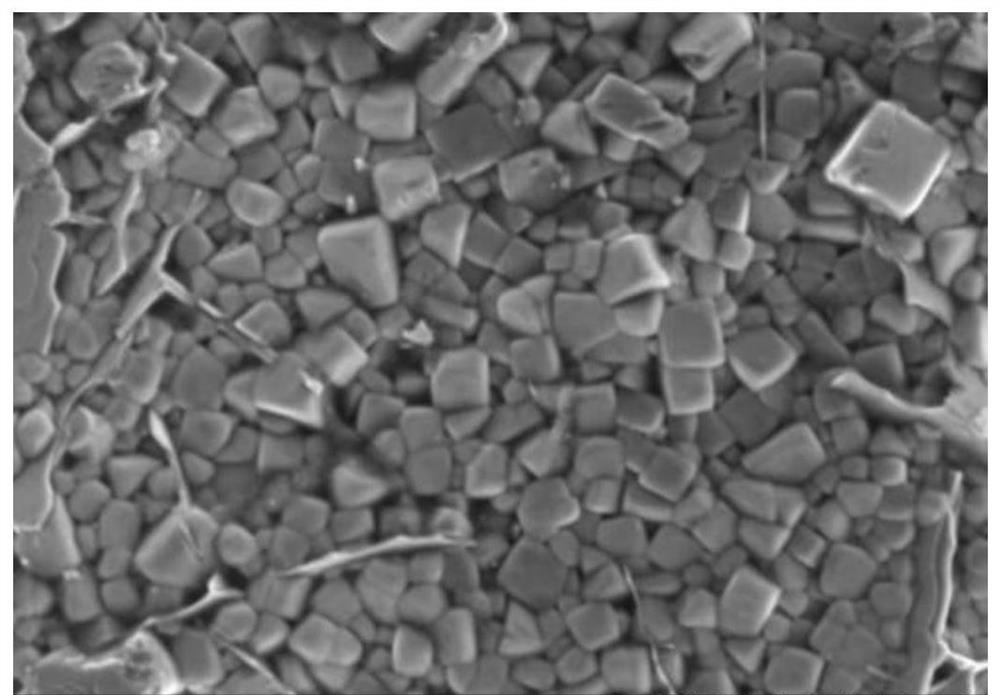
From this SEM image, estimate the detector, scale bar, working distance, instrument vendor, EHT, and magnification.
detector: SE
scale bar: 10000 nm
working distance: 15.1 mm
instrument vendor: Hitachi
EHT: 10 kV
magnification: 4 K X